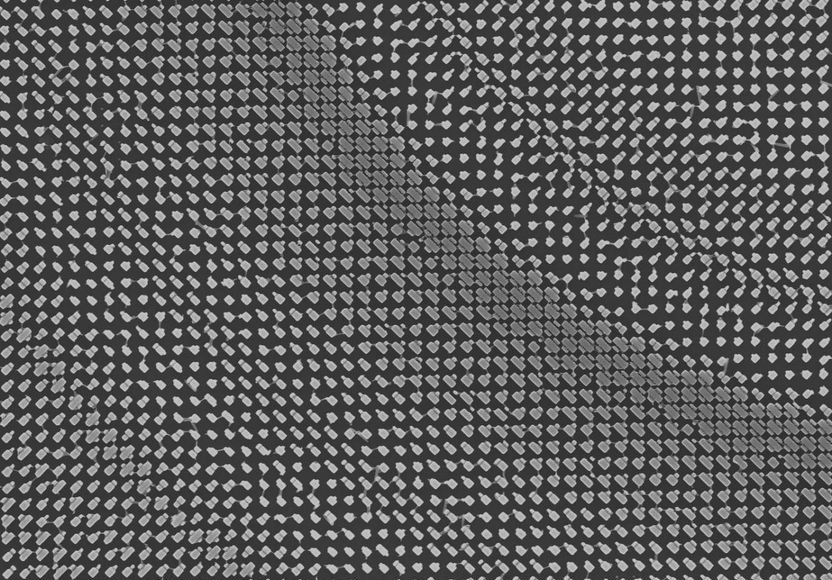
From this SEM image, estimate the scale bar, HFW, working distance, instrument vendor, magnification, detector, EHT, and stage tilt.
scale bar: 5000 nm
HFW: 20.7 µm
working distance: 4 mm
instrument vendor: FEI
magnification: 10 K X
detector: TLD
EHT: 20 kV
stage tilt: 0°